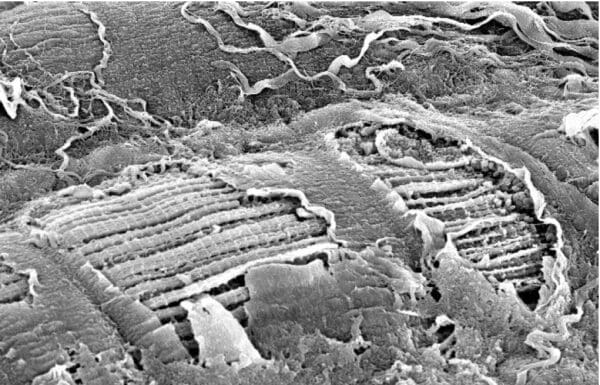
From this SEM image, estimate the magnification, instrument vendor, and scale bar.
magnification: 2 K X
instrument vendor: FEI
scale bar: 10000 nm